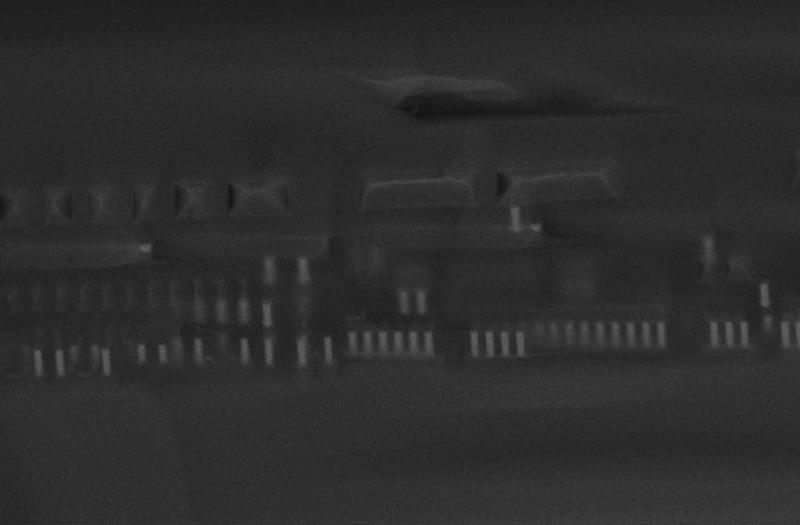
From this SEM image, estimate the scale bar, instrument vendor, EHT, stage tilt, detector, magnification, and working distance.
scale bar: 2000 nm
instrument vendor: Zeiss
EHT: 15 kV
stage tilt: -15°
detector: InLens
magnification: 11.47 K X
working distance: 4 mm